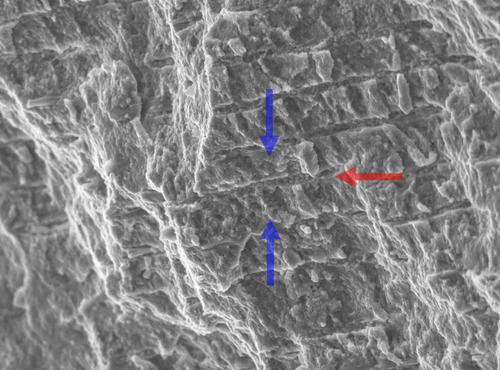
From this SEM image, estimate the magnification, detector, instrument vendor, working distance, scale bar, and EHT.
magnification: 2 K X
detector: SED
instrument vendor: JEOL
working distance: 13.3 mm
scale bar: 10000 nm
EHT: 10 kV